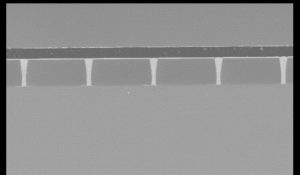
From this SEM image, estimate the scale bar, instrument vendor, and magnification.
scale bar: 500000 nm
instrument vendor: Hitachi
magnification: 0.11 K X